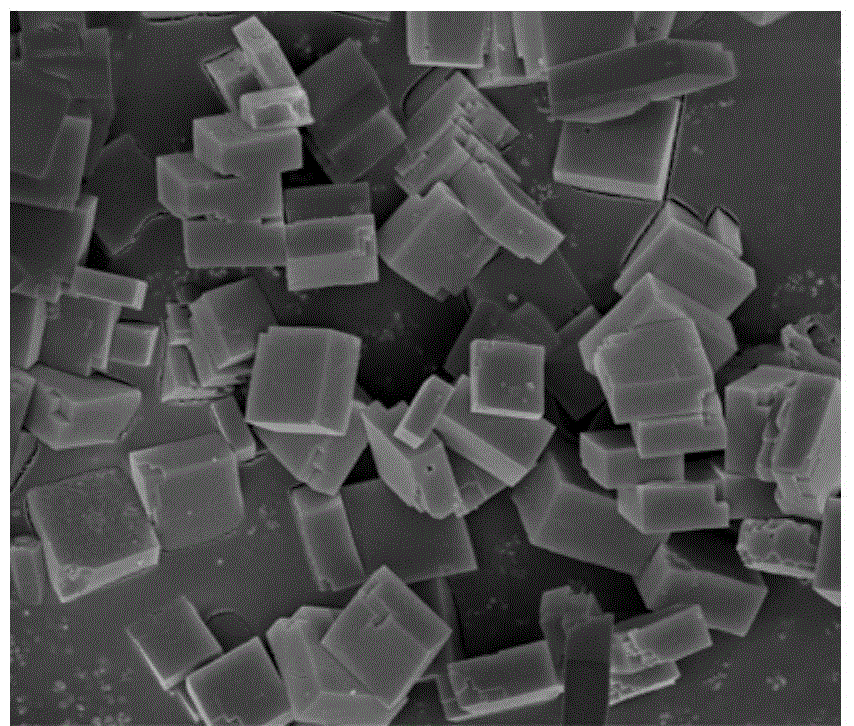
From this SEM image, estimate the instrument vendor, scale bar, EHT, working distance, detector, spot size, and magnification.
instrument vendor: FEI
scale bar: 5000 nm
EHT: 5 kV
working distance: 4.7 mm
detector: TLD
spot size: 3.5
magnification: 20 K X